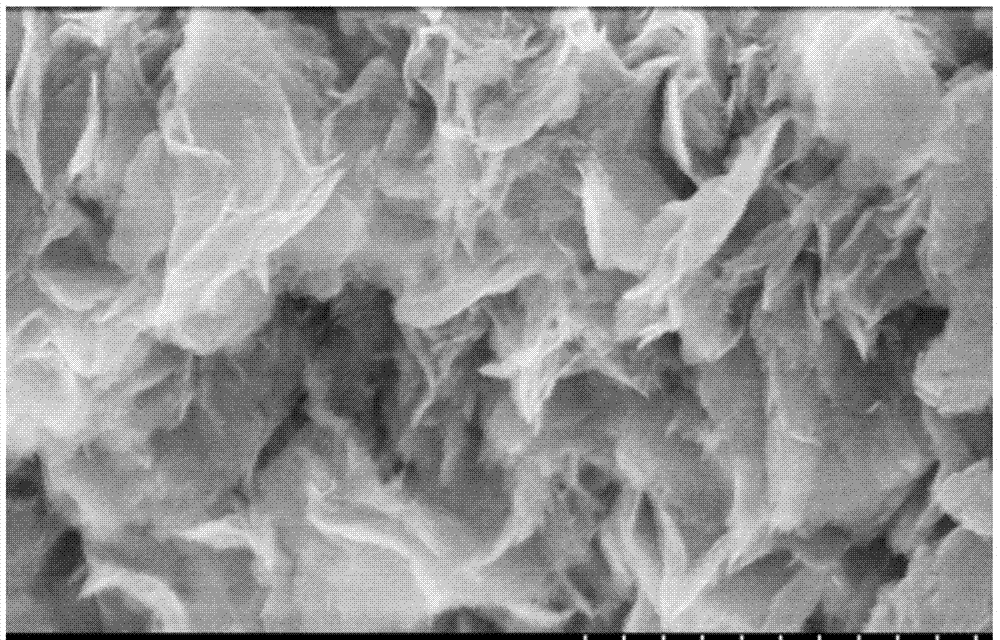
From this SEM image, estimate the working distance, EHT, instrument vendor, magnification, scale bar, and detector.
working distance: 7.7 mm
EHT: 10 kV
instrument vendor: Hitachi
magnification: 50 K X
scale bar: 1000 nm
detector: SE(M)